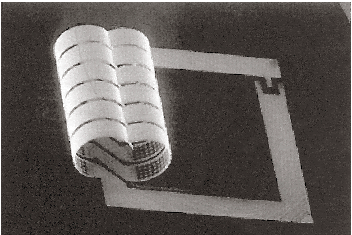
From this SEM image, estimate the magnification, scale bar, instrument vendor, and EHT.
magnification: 0.0292 K X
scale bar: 1000000 nm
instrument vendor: other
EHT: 20 kV